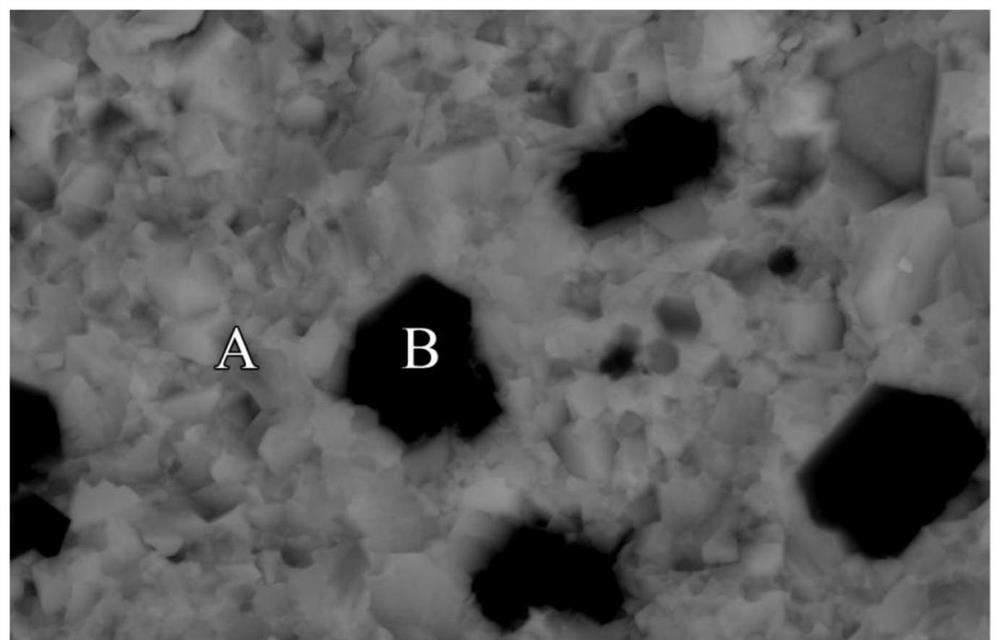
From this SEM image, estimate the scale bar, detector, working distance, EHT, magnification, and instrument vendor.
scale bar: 5000 nm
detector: BSE-ALL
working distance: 11.3 mm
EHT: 20 kV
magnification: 10 K X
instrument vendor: Hitachi